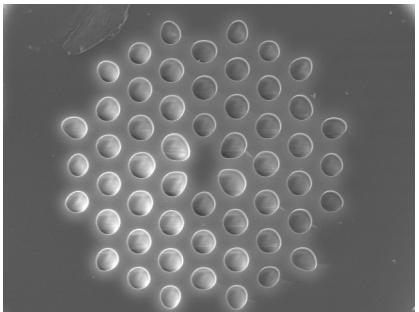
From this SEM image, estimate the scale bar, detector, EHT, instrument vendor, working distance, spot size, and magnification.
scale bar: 20000 nm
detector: SE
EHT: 20 kV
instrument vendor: FEI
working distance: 7.8 mm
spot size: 4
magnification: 3 K X